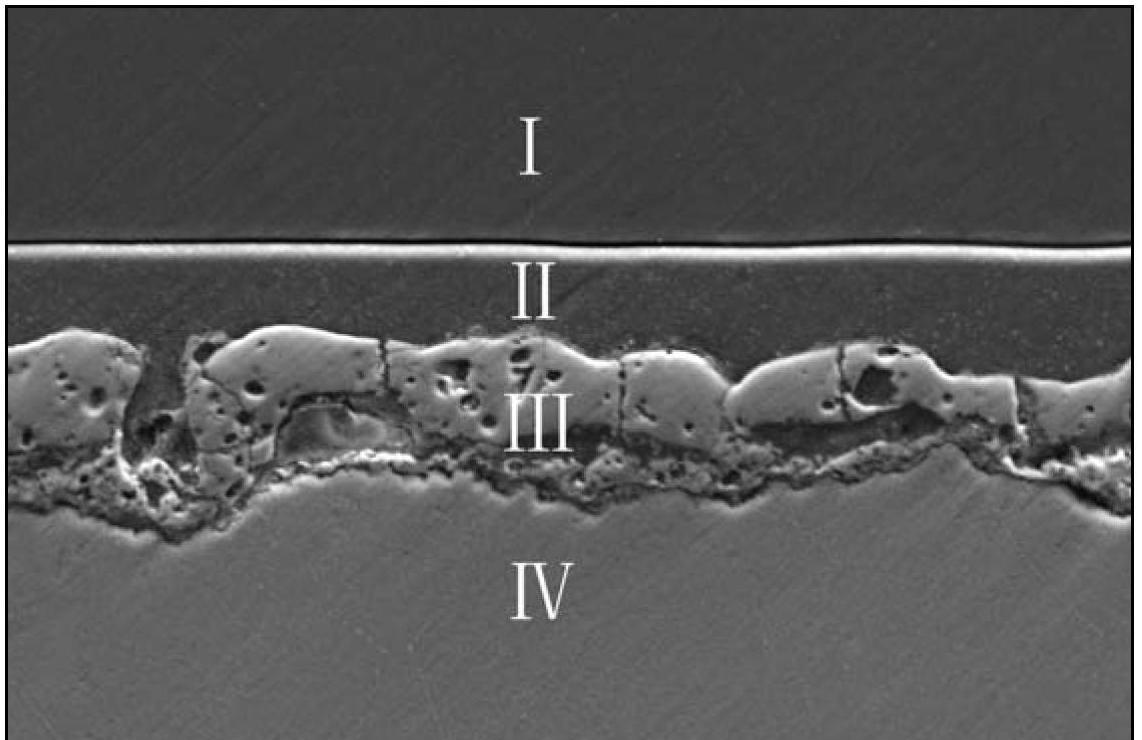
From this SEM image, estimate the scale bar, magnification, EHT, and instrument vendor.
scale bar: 50000 nm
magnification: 0.5 K X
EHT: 20 kV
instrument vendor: JEOL